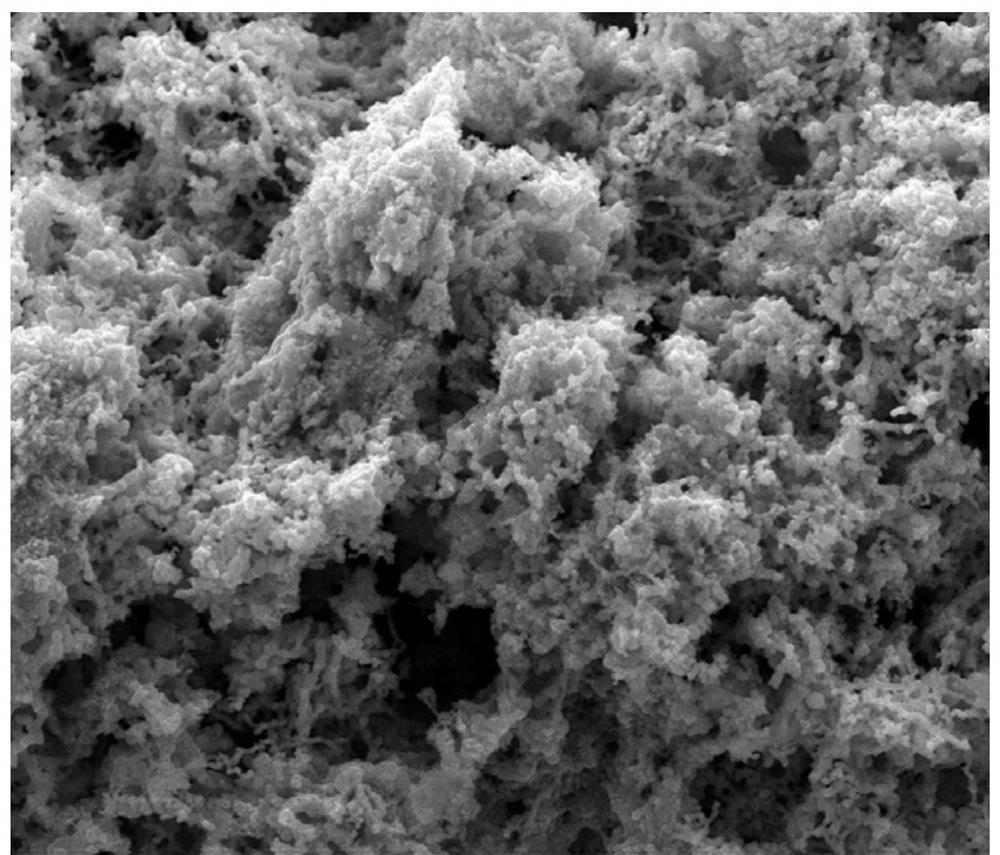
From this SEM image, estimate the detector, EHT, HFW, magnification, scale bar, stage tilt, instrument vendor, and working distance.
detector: ETD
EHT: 20 kV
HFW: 29.8 µm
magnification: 10 K X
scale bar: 10000 nm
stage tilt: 0°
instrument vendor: FEI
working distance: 9 mm